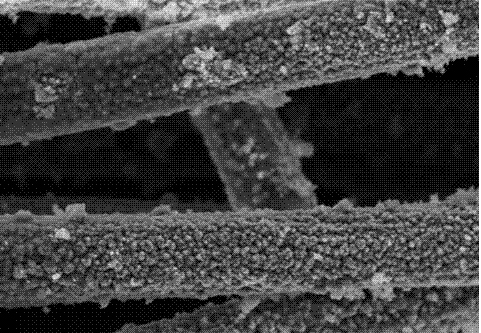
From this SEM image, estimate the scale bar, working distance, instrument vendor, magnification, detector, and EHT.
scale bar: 10000 nm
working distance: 8.3 mm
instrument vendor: Hitachi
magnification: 3 K X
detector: SE(U)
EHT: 10 kV